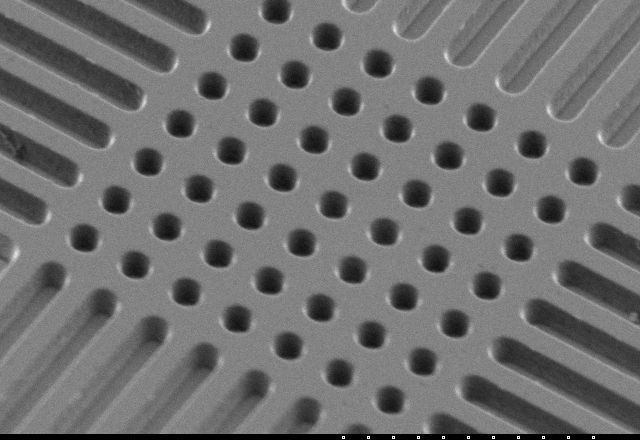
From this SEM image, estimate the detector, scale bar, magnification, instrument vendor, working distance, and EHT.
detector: SE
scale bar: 10000 nm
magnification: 5 K X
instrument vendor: Hitachi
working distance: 11.9 mm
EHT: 5 kV